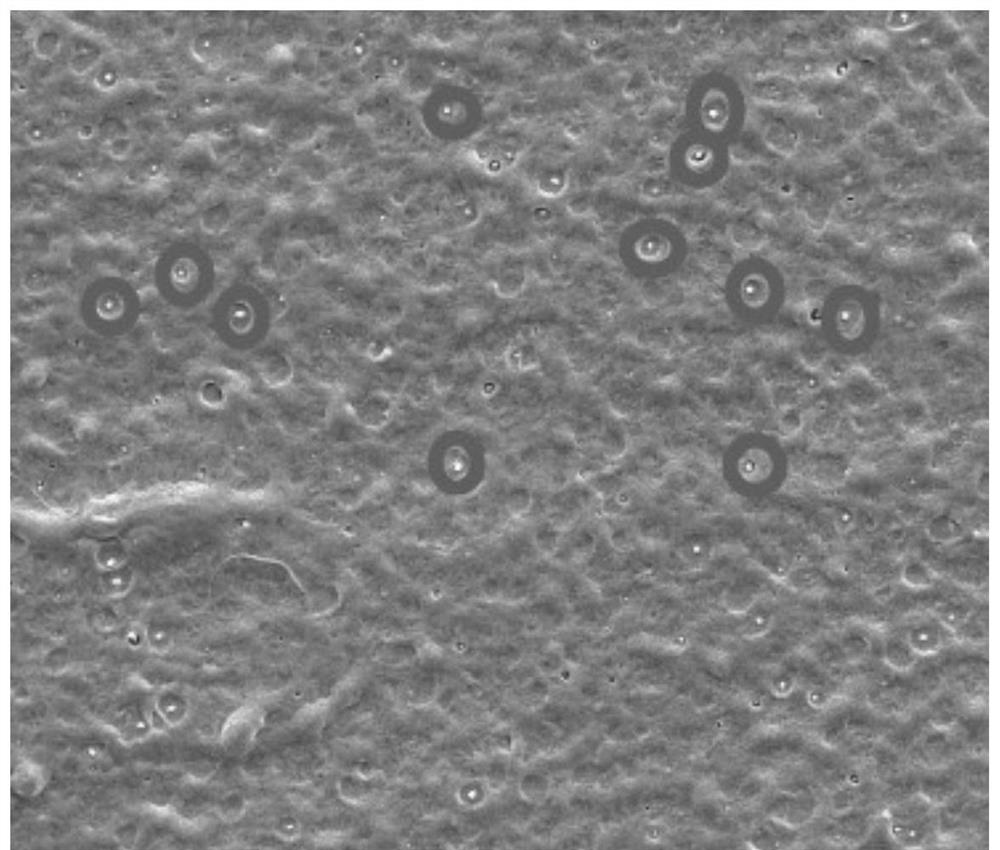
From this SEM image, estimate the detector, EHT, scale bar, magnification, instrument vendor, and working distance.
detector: ETD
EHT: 10 kV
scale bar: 10000 nm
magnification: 10 K X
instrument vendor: FEI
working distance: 10.2 mm